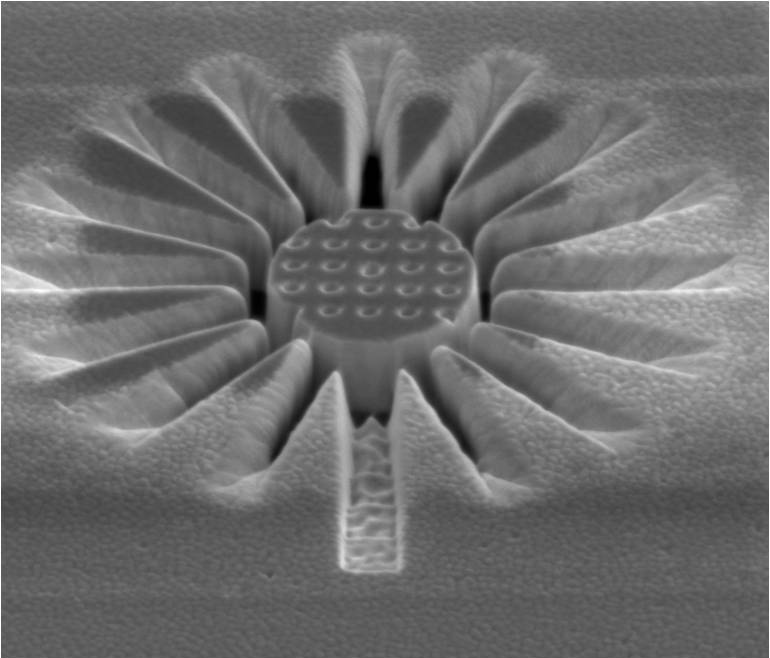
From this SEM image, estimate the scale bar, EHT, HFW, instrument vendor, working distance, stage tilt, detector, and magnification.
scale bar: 1000 nm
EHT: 5 kV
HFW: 5.97 µm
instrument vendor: FEI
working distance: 4.1 mm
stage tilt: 50°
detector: ETD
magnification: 50 K X